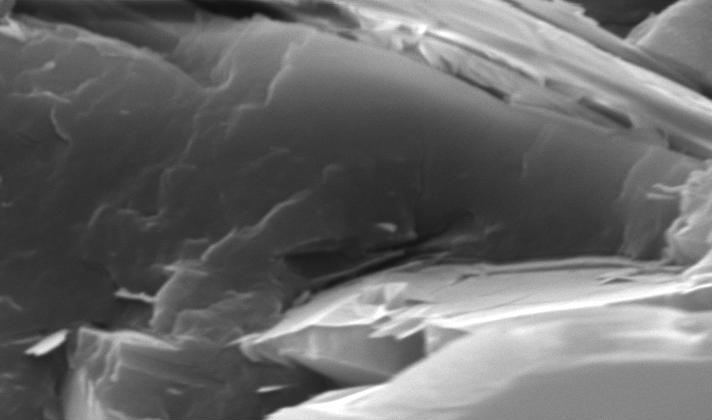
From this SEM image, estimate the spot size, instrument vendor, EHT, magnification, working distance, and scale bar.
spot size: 5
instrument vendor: FEI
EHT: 20 kV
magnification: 4 K X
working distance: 6.2 mm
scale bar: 5000 nm